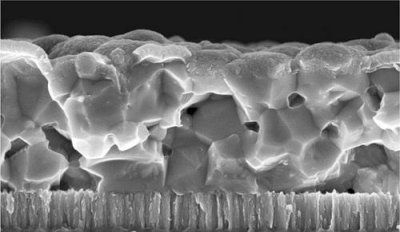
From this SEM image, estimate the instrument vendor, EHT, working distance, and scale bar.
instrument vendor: Zeiss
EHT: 10 kV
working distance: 8 mm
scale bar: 1000 nm